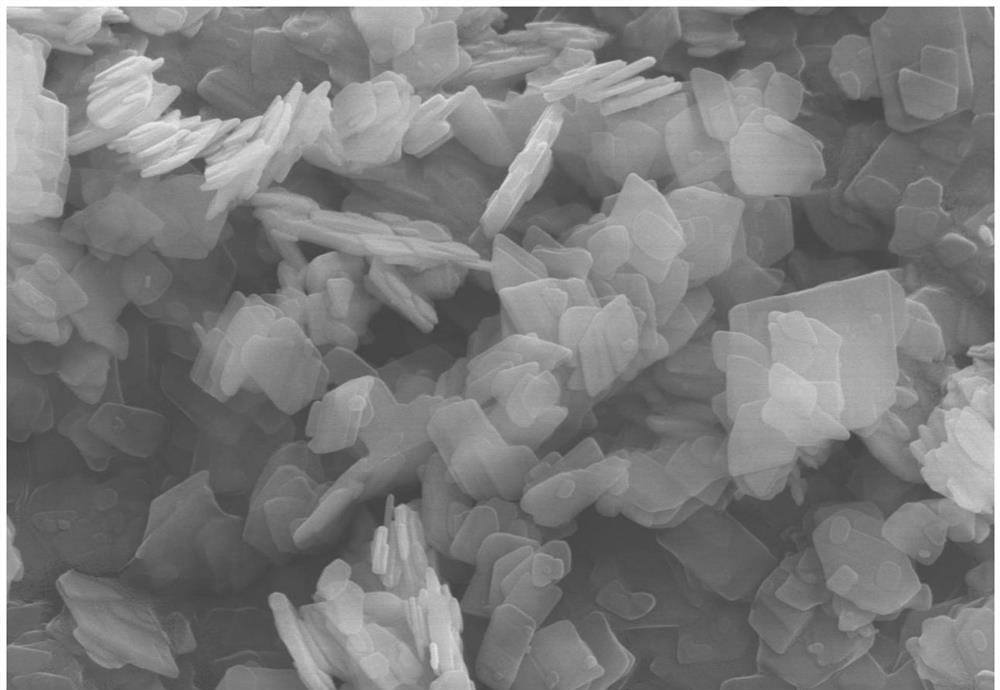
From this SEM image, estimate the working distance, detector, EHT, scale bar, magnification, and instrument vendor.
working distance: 8.2 mm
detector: SE(UL)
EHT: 15 kV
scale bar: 3000 nm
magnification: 15 K X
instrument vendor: Hitachi